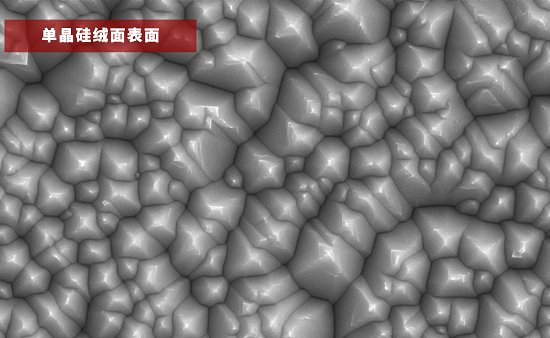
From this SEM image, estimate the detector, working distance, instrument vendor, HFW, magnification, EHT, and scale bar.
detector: SED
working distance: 9.521 mm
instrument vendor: other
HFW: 12.7 µm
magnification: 10 K X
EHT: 10 kV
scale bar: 3000 nm